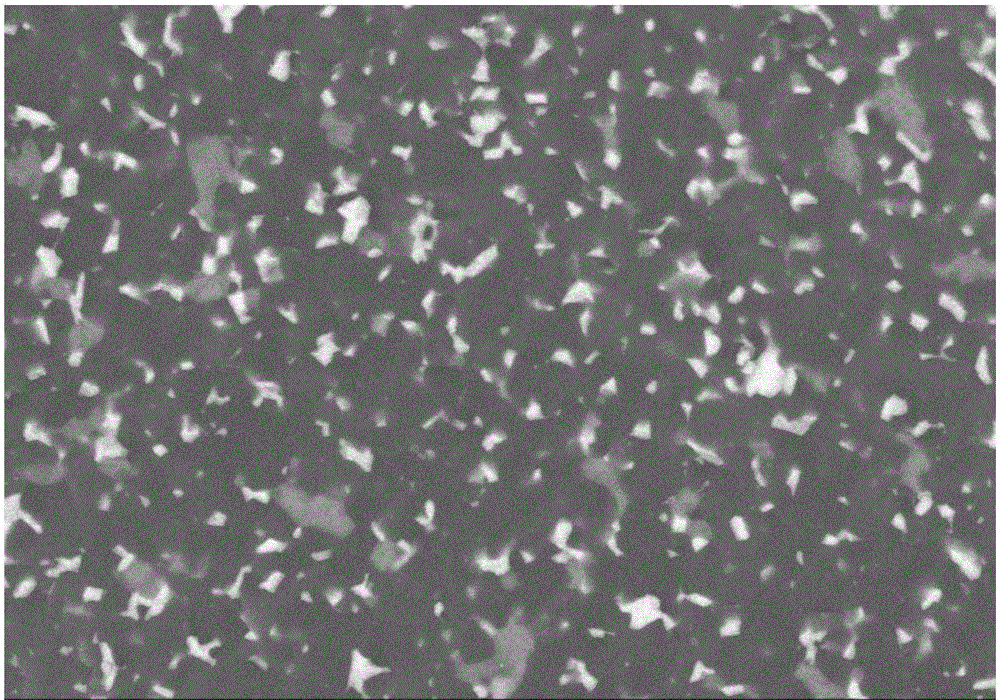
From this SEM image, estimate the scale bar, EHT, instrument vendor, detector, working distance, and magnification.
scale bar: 20000 nm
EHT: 15 kV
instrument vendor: Hitachi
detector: BSE3D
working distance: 13.2 mm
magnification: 2 K X